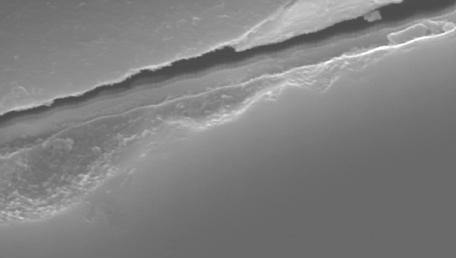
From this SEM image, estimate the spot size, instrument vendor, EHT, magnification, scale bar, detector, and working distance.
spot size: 3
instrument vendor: FEI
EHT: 20 kV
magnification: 10 K X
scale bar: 2000 nm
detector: SE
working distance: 11.1 mm